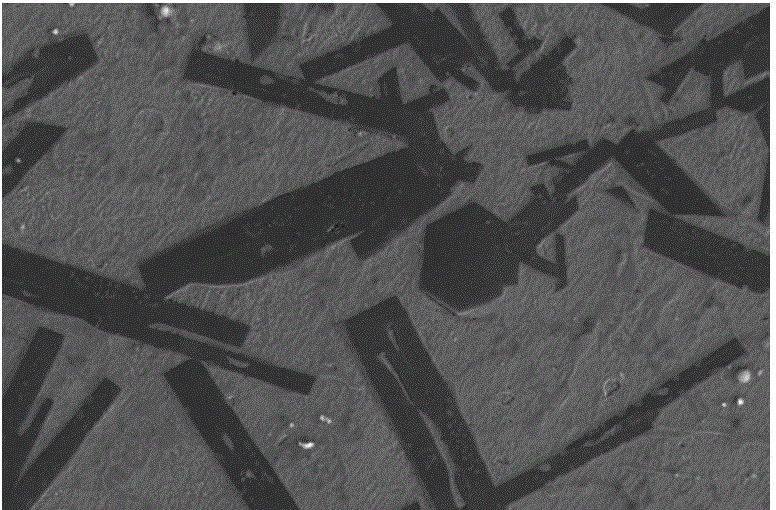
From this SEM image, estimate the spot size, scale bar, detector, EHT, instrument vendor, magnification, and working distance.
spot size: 5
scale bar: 10000 nm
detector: ETD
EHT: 10 kV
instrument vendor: FEI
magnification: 5 K X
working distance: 5.1 mm